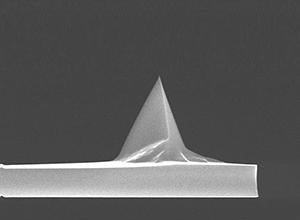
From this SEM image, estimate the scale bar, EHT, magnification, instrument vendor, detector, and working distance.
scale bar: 10000 nm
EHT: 15 kV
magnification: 3.5 K X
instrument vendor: FEI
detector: TLD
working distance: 5.5 mm